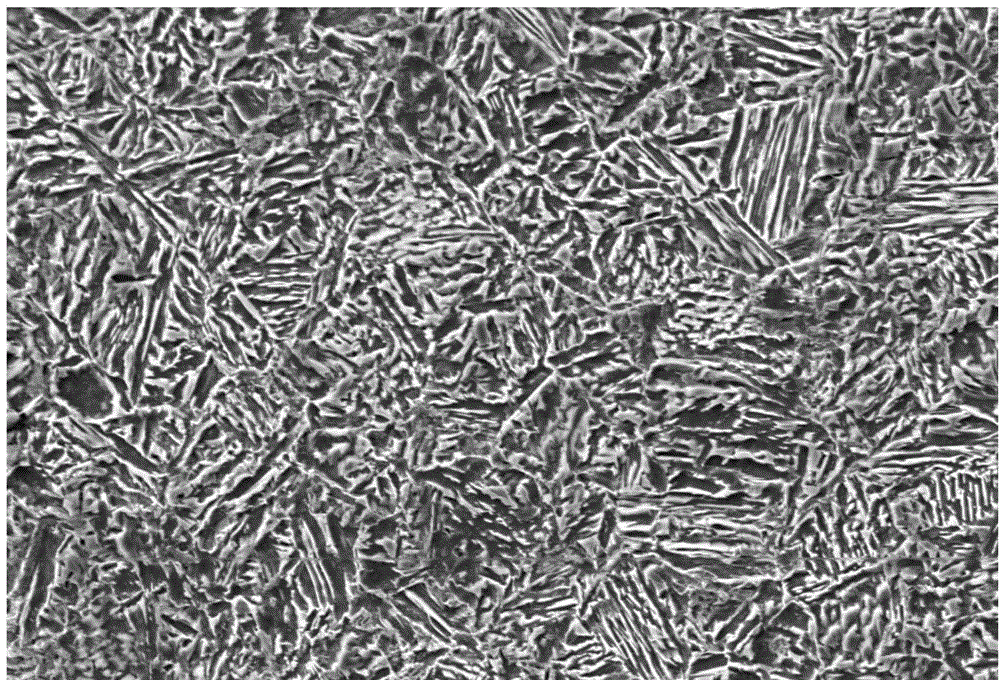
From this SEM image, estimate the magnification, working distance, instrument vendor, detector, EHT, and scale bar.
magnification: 1 K X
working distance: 10 mm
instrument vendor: Zeiss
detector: SE1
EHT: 20 kV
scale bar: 10000 nm